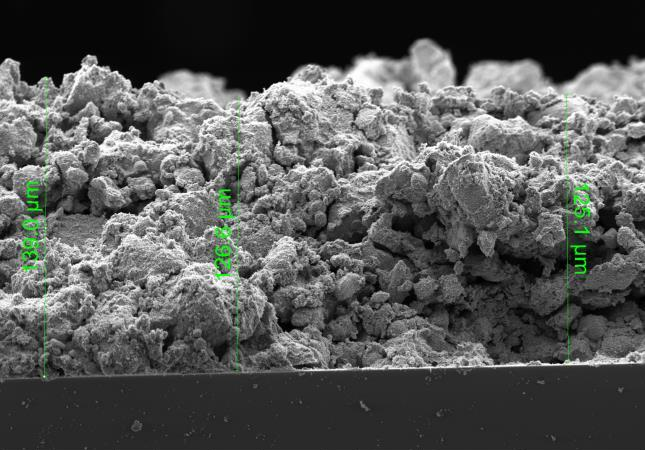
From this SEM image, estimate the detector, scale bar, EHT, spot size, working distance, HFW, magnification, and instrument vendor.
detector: ETD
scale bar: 50000 nm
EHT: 5 kV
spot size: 3.5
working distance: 10.4 mm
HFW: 317 µm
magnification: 1.309 K X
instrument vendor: FEI